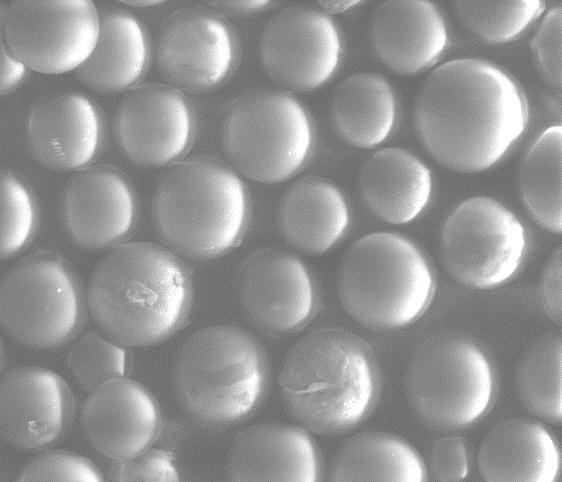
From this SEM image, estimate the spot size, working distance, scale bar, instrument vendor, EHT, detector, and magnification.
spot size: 5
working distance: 10.9 mm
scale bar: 100000 nm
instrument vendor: FEI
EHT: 15 kV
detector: LFD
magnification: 0.4 K X